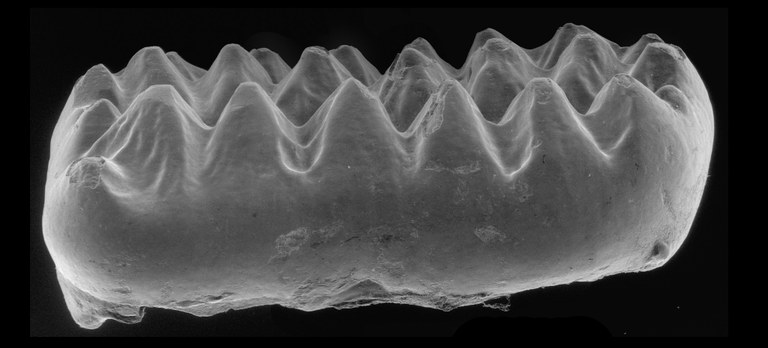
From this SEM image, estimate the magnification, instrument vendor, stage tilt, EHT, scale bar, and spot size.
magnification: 0.015 K X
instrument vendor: FEI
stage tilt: -15°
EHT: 20 kV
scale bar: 10000000 nm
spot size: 4